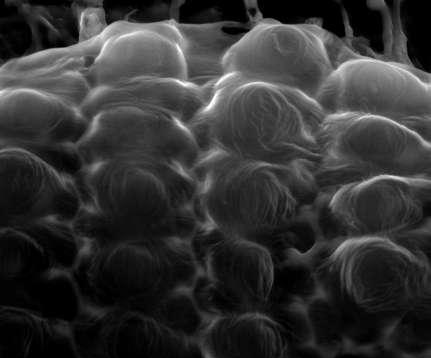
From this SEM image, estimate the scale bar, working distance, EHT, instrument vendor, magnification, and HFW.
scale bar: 20000 nm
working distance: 8.2 mm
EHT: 15 kV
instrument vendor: FEI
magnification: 4 K X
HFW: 67.6 µm